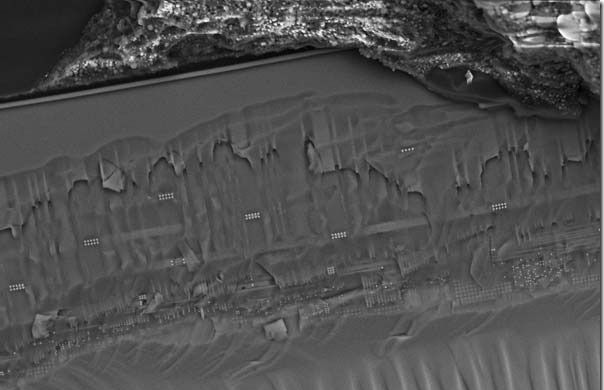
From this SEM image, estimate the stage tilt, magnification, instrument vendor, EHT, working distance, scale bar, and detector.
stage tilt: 46.8°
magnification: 1.82 K X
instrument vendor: Zeiss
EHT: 15 kV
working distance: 2.5 mm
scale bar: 10000 nm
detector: InLens